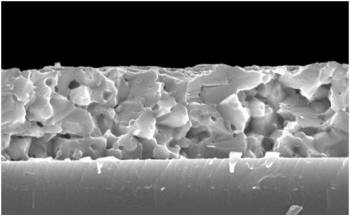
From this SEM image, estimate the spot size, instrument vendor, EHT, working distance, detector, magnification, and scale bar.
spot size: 3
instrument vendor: FEI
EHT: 10 kV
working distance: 6.5 mm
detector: TLD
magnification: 20 K X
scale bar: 1000 nm